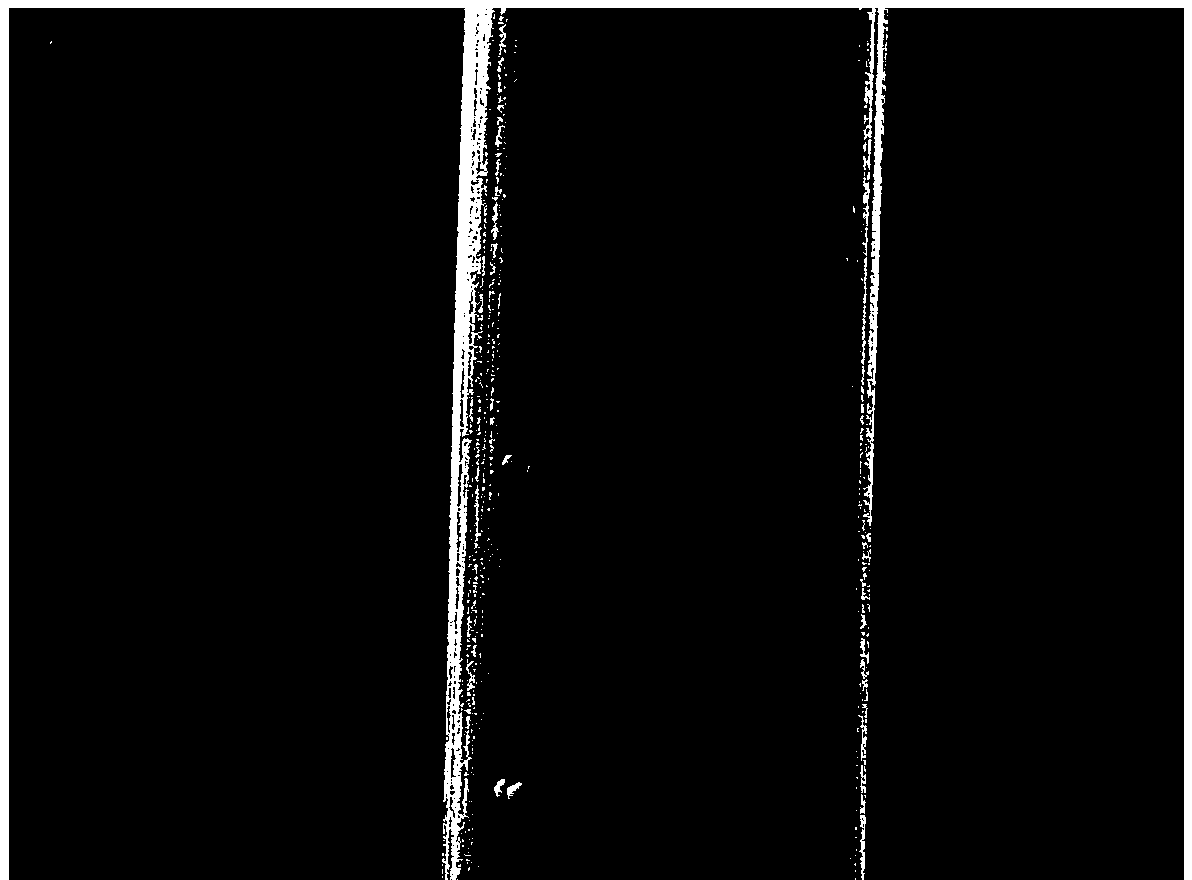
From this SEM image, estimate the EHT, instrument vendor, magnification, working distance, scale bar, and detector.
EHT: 20 kV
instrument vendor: Tescan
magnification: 10 K X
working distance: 15.9 mm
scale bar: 5000 nm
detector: SE